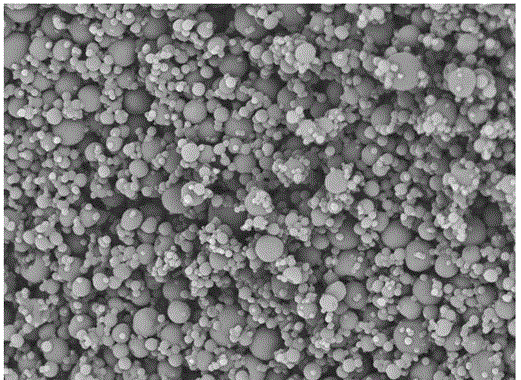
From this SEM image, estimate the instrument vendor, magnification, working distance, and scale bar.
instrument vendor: Hitachi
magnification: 2 K X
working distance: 4.9 mm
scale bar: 30000 nm